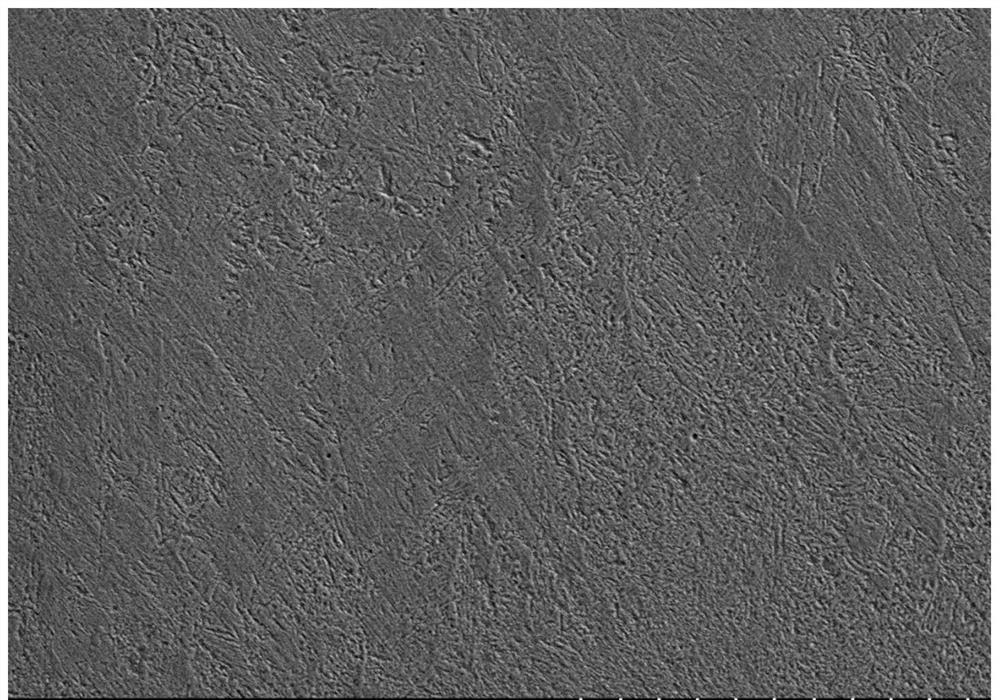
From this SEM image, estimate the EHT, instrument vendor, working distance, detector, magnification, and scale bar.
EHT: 15 kV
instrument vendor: Hitachi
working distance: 8.7 mm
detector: SE(L)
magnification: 1 K X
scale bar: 50000 nm